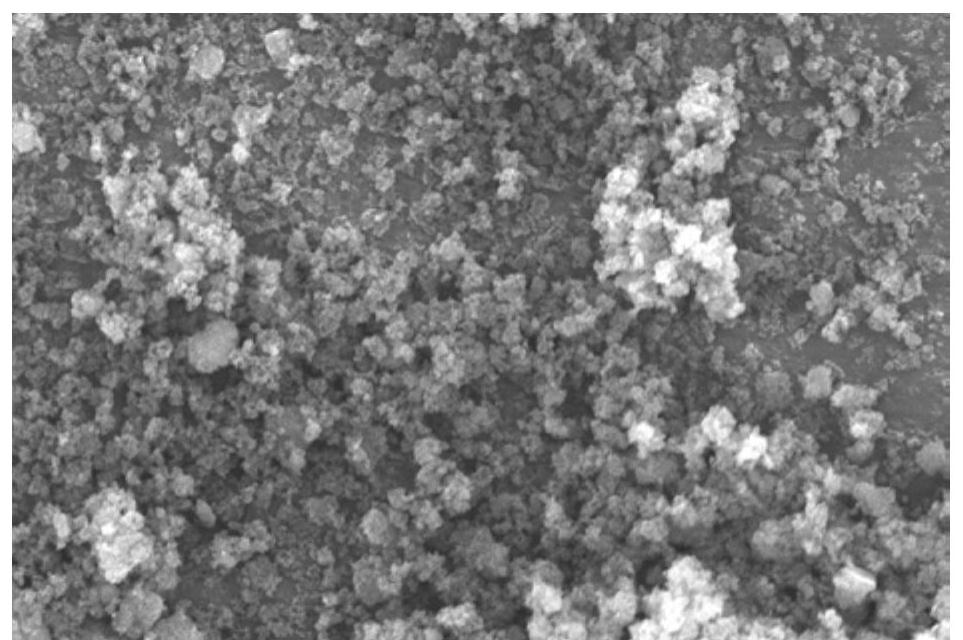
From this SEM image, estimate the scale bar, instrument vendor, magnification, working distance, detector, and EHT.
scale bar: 5000 nm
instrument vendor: JEOL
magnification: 3 K X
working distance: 10 mm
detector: SEI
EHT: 20 kV